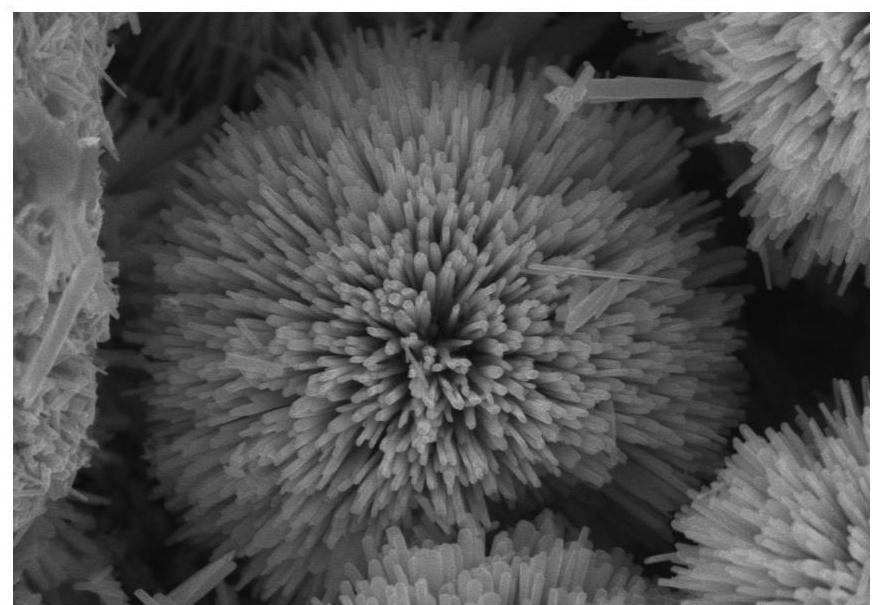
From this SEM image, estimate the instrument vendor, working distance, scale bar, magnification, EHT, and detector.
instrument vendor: Hitachi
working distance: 8.3 mm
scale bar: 1000 nm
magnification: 35 K X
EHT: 5 kV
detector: SE(U)